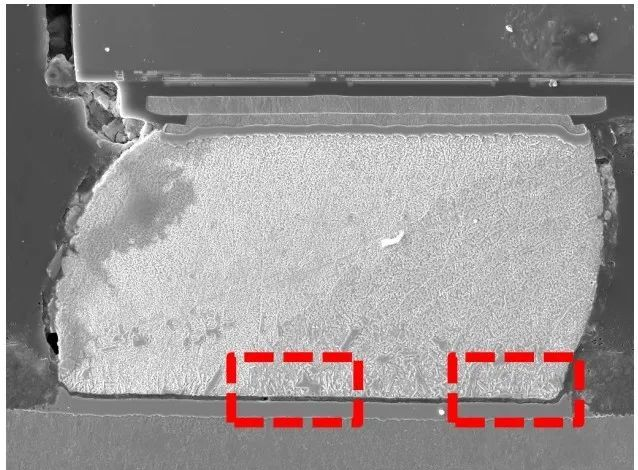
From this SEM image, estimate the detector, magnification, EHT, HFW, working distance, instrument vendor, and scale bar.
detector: SE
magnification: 1.24 K X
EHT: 20 kV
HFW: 224 µm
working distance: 19.97 mm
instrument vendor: Tescan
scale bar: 50000 nm